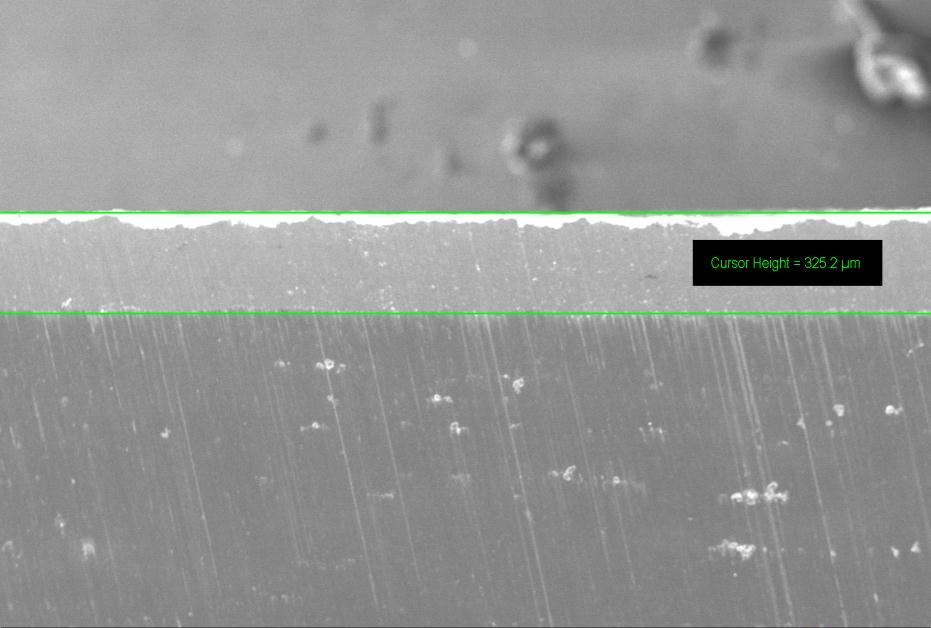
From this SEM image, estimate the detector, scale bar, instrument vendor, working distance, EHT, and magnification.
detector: SE1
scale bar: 100000 nm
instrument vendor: Zeiss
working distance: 30.5 mm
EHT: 20 kV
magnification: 0.1 K X